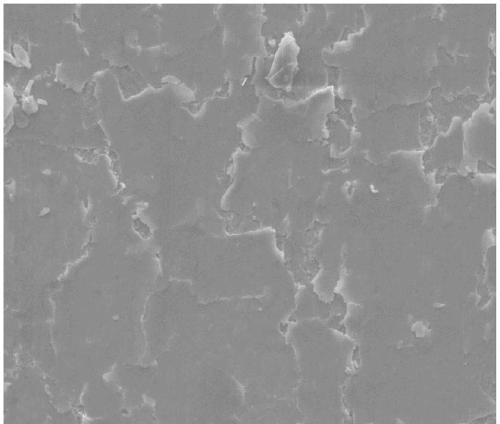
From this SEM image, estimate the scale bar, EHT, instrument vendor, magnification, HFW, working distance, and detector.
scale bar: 50000 nm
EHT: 20 kV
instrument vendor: FEI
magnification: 0.5 K X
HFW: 270 µm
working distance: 10.9 mm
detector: ETD